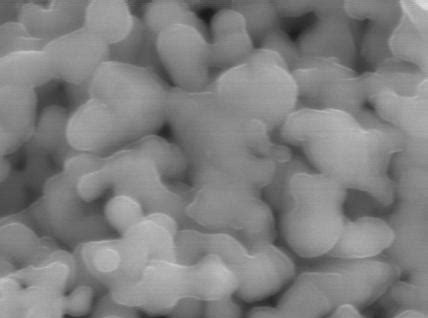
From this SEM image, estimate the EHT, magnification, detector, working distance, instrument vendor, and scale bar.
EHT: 10 kV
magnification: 75 K X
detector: SEI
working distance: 16.1 mm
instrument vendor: JEOL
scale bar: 100 nm